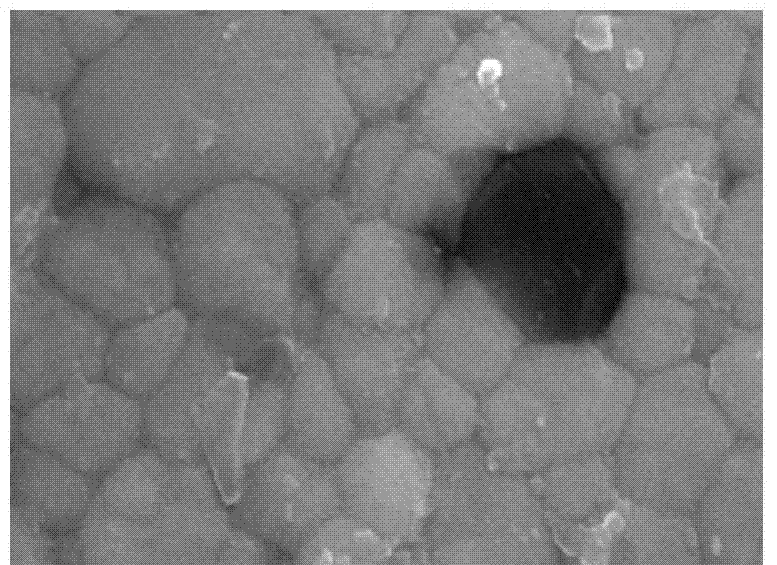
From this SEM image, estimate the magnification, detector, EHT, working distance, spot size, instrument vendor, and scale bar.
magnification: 40 K X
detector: SE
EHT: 20 kV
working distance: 6.3 mm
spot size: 4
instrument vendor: FEI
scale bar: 500 nm